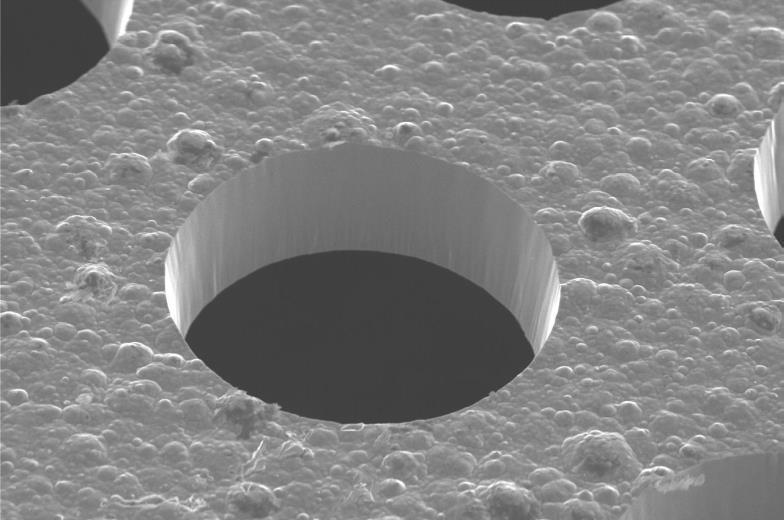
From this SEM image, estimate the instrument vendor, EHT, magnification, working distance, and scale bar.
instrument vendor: Hitachi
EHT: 20 kV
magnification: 0.5 K X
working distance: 20.5 mm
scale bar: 20000 nm